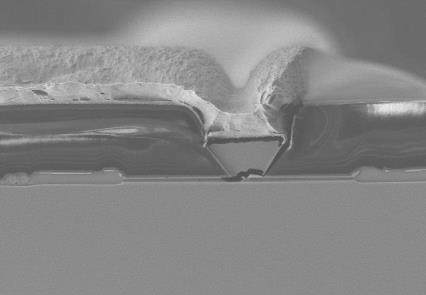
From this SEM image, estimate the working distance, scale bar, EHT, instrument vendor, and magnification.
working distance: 12.3 mm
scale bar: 10000 nm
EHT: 5 kV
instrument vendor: Hitachi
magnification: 5 K X